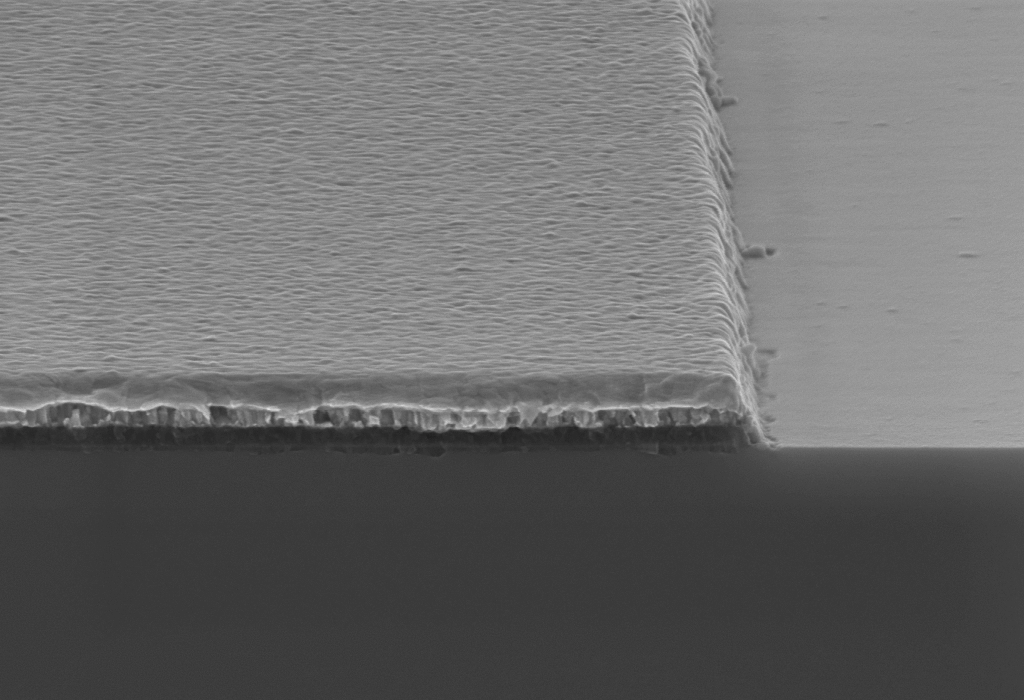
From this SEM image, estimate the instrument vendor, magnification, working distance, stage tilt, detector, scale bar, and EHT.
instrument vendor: Zeiss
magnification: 100 K X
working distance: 3.7 mm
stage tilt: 3.9°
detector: InLens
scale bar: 200 nm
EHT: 7 kV